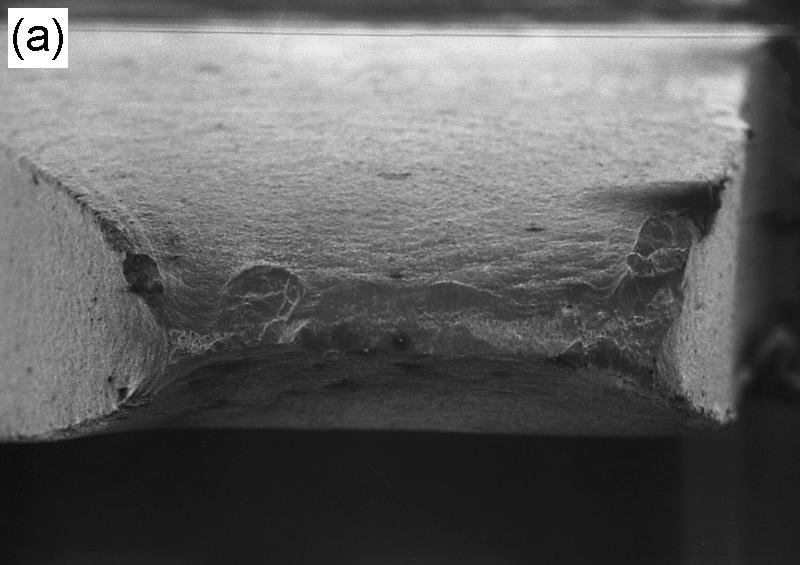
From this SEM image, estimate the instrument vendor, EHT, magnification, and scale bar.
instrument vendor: JEOL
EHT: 20 kV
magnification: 0.0204 K X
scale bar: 1000000 nm